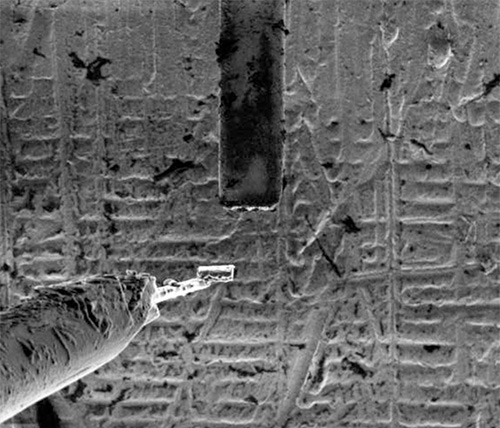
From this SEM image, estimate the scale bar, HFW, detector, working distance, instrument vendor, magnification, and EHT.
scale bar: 50000 nm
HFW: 304 µm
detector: SED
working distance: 18 mm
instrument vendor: FEI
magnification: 1 K X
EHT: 30 kV